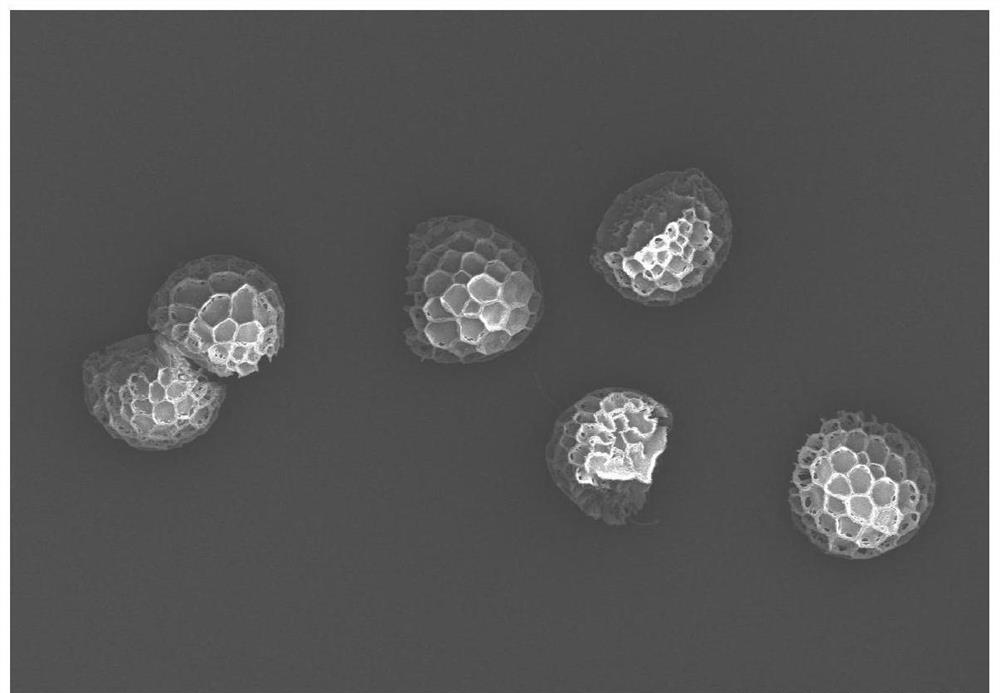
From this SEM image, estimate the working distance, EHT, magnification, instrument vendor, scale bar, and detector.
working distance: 8.4 mm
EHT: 3 kV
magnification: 0.6 K X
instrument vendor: Hitachi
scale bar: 50000 nm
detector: SE(U)